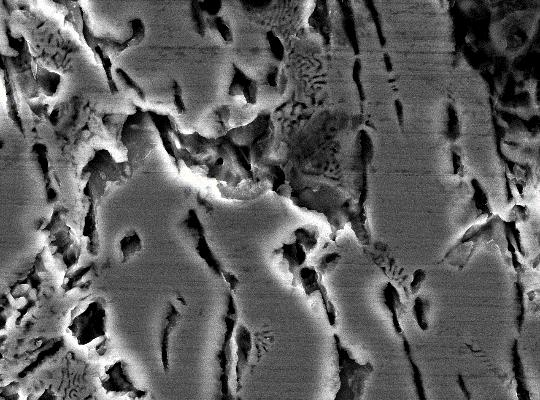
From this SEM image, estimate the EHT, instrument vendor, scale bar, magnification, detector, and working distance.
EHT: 15 kV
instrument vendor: JEOL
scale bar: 1000 nm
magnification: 5 K X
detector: SEI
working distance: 10.2 mm